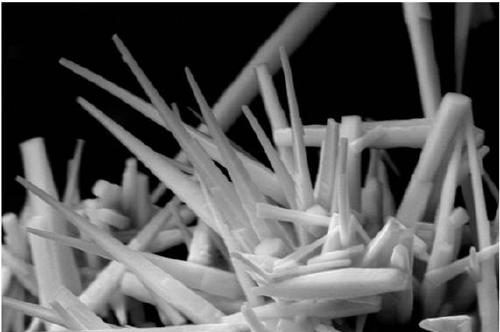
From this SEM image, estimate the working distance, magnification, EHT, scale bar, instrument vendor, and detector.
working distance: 9.8 mm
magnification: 10 K X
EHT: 15 kV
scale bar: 1000 nm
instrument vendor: Zeiss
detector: SE2